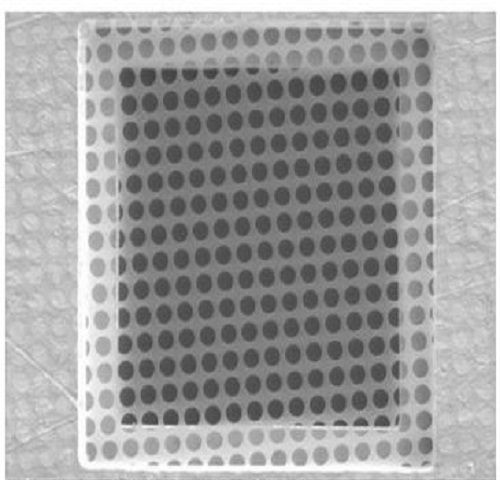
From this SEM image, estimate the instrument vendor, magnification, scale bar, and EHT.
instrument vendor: other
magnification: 1.65 K X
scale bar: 20000 nm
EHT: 5 kV